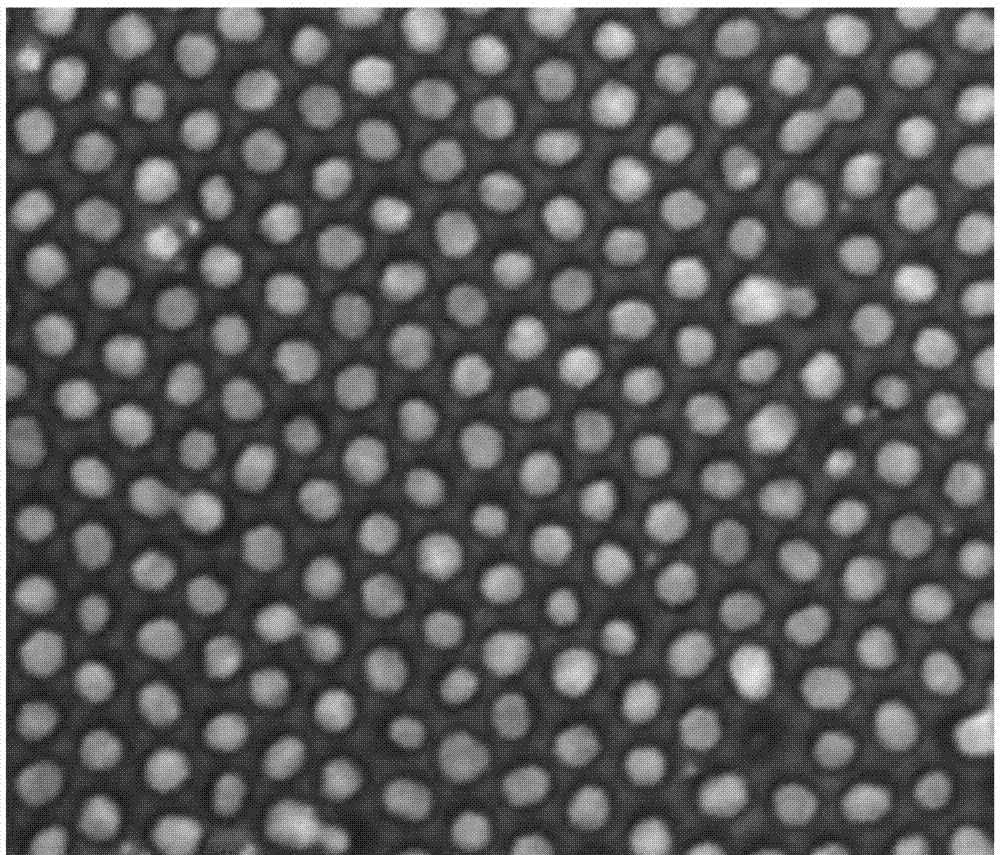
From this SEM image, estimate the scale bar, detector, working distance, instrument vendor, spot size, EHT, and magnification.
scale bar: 500 nm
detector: ETD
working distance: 10.1 mm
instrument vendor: FEI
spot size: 3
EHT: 20 kV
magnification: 200 K X